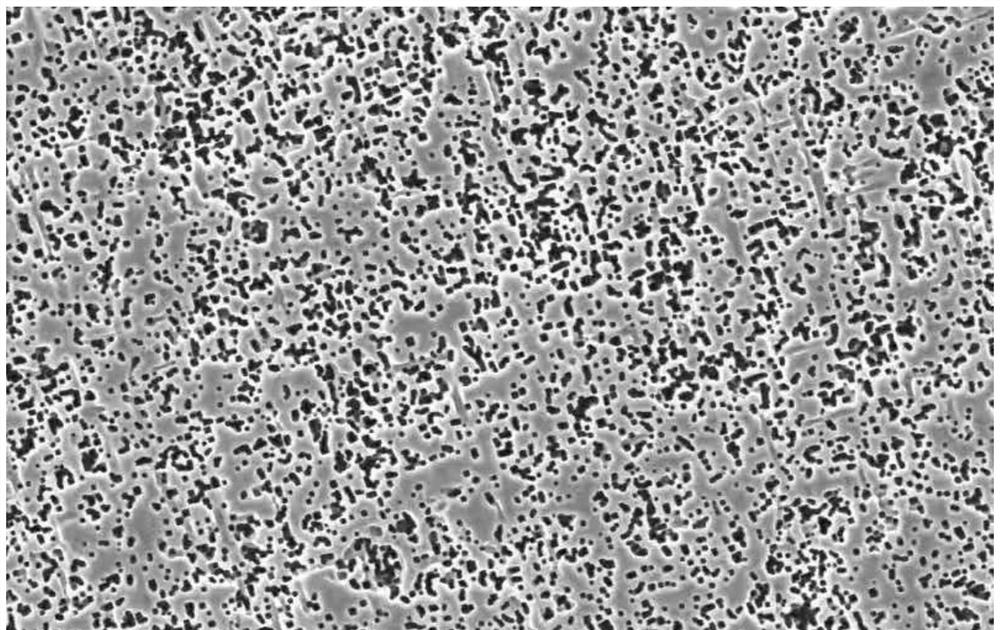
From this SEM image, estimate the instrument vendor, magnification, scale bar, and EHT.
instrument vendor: other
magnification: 0.8 K X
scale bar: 100000 nm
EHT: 10 kV